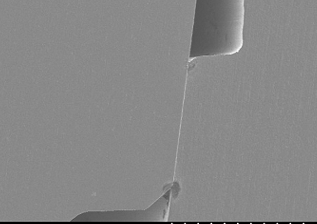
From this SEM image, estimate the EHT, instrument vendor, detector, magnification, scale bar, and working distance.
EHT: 10 kV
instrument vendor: Hitachi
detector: SE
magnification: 0.1 K X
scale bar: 500000 nm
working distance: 15.1 mm